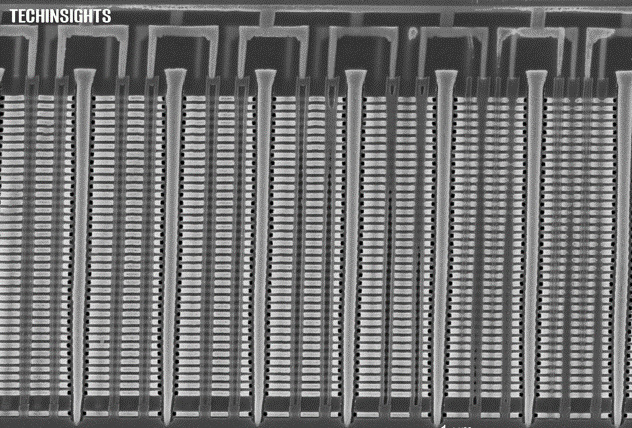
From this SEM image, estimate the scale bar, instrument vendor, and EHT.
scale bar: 1000 nm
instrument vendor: Zeiss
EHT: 5 kV